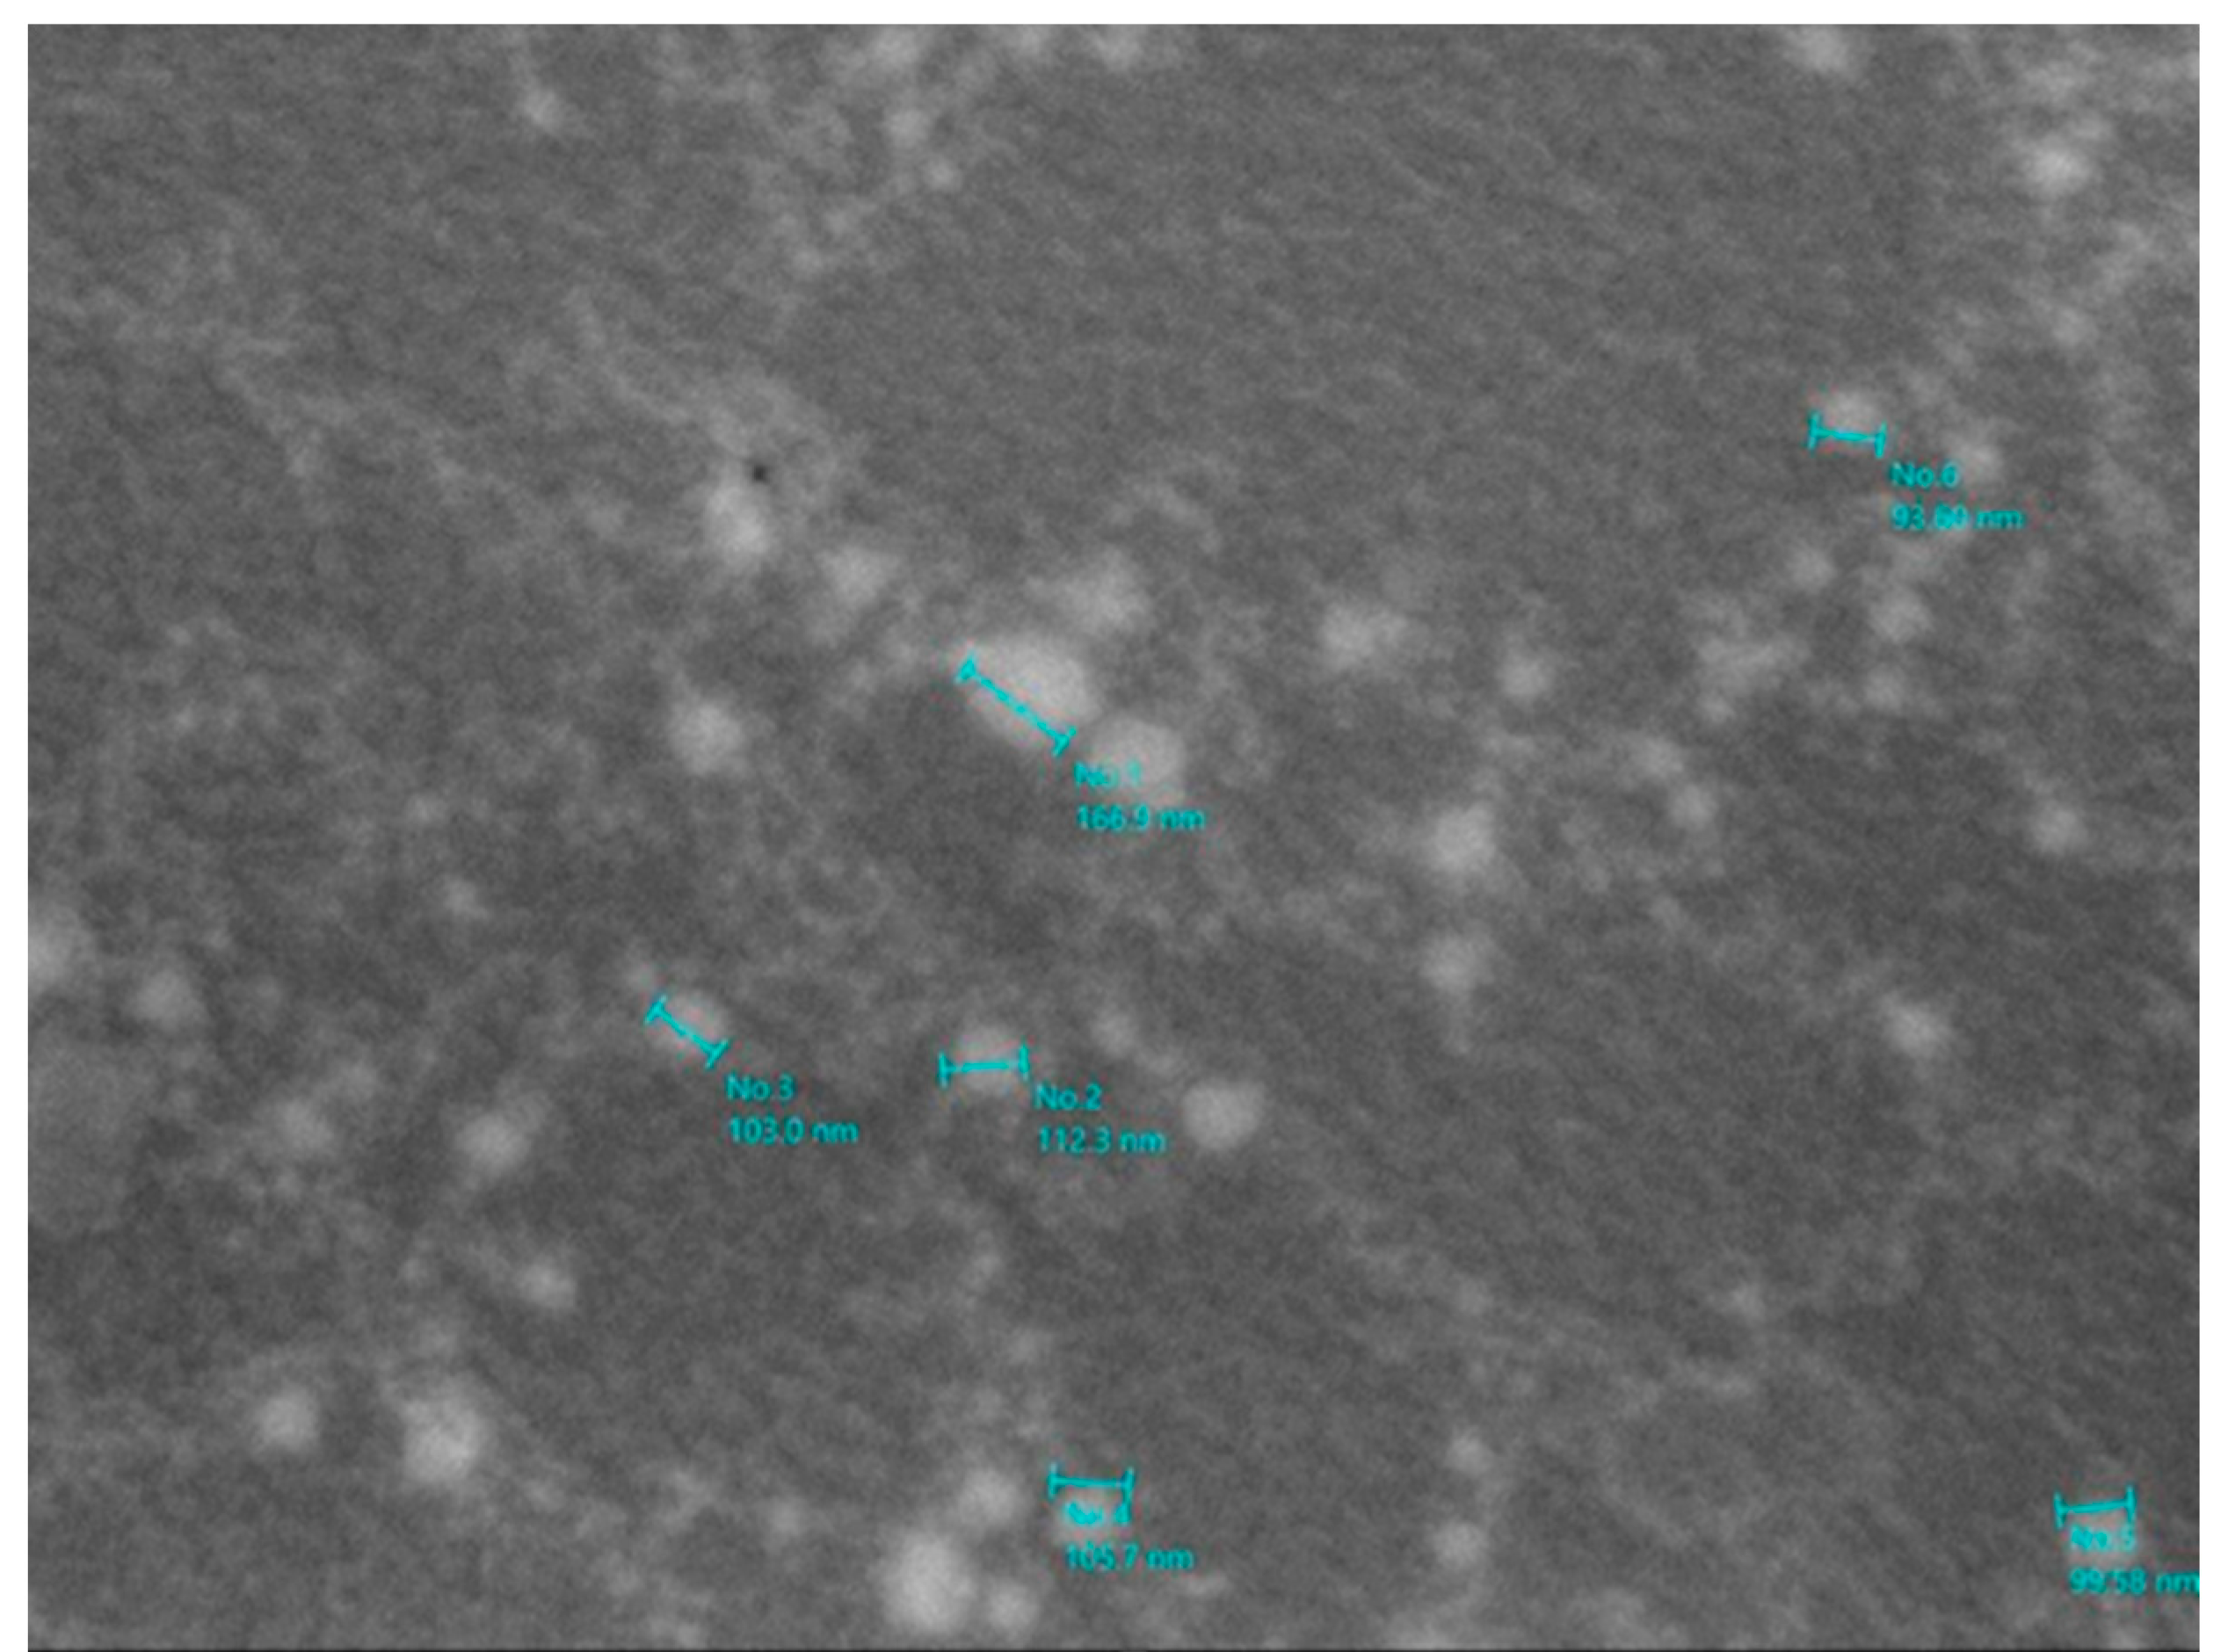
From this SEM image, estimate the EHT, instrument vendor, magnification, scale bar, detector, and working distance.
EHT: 10 kV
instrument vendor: JEOL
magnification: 43 K X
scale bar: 500 nm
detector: SED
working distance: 10.5 mm